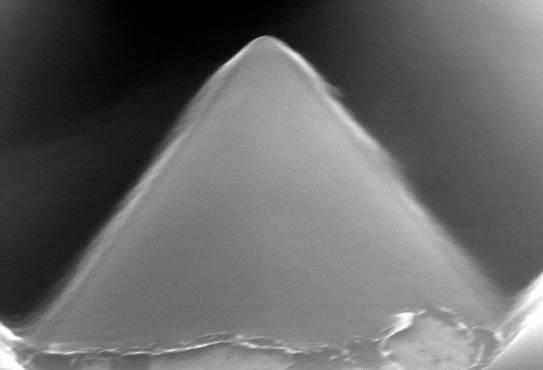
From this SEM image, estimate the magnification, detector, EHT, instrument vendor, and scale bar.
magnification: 40 K X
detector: SE(TUL)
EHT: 15 kV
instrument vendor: Hitachi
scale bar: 1000 nm